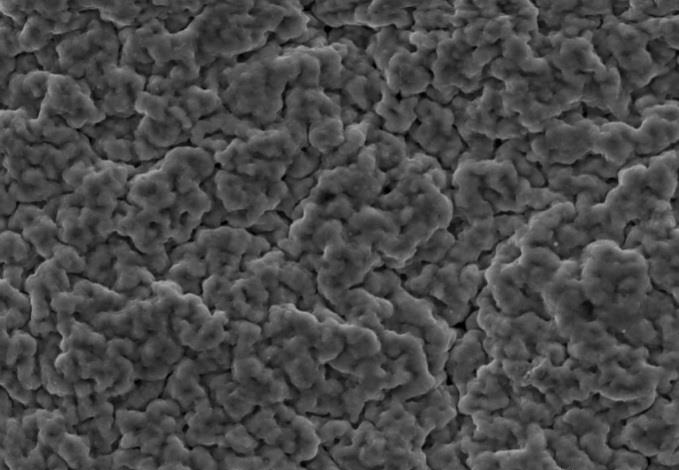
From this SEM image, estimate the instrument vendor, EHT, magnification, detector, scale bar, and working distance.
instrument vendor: Tescan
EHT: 20 kV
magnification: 2 K X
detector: SE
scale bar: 20000 nm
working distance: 15 mm